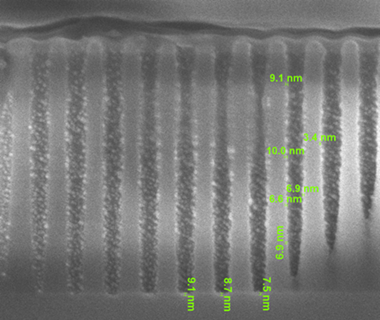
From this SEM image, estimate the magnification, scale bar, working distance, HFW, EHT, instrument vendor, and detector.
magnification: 100 K X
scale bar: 500 nm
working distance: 3.5 mm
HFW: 1.28 µm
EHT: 3 kV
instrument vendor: FEI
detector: SE